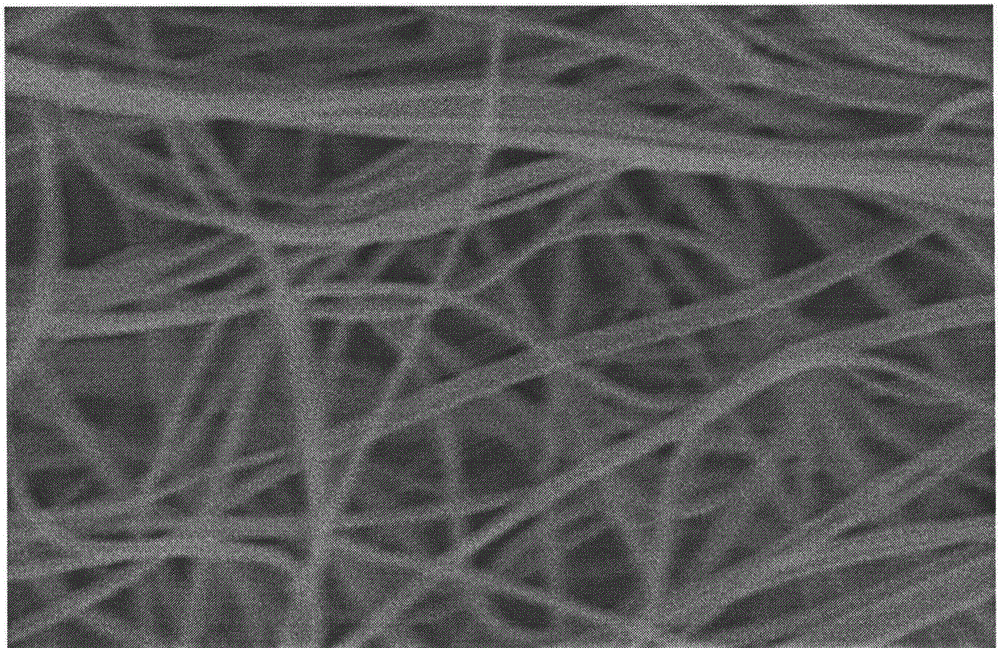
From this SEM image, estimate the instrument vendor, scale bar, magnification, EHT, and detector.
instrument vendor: Hitachi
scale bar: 5000 nm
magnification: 10 K X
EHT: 10 kV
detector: SE(M)